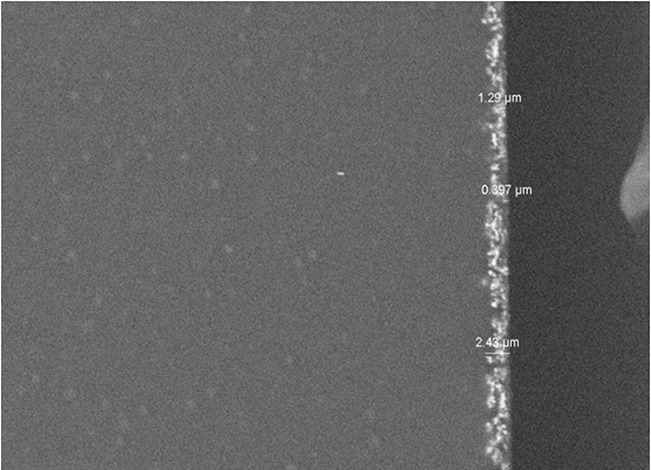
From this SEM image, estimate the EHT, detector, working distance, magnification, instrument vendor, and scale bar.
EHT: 10 kV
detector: BSE3D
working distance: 10.2 mm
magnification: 2 K X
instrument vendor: Hitachi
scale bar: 20000 nm